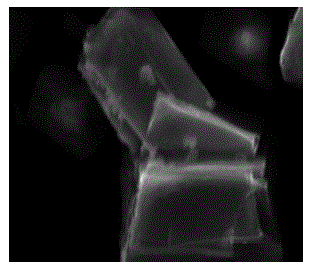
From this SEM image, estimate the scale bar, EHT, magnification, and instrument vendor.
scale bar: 5000 nm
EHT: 15 kV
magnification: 5 K X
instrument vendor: JEOL